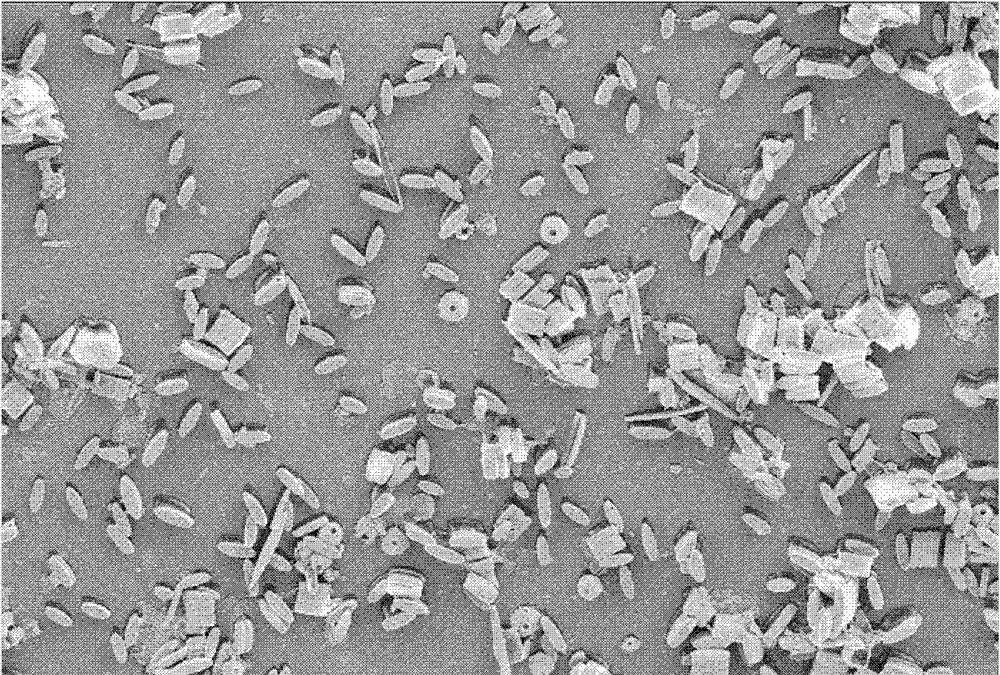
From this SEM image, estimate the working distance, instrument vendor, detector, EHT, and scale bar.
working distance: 6 mm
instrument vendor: Zeiss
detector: SE2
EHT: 5 kV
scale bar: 20000 nm